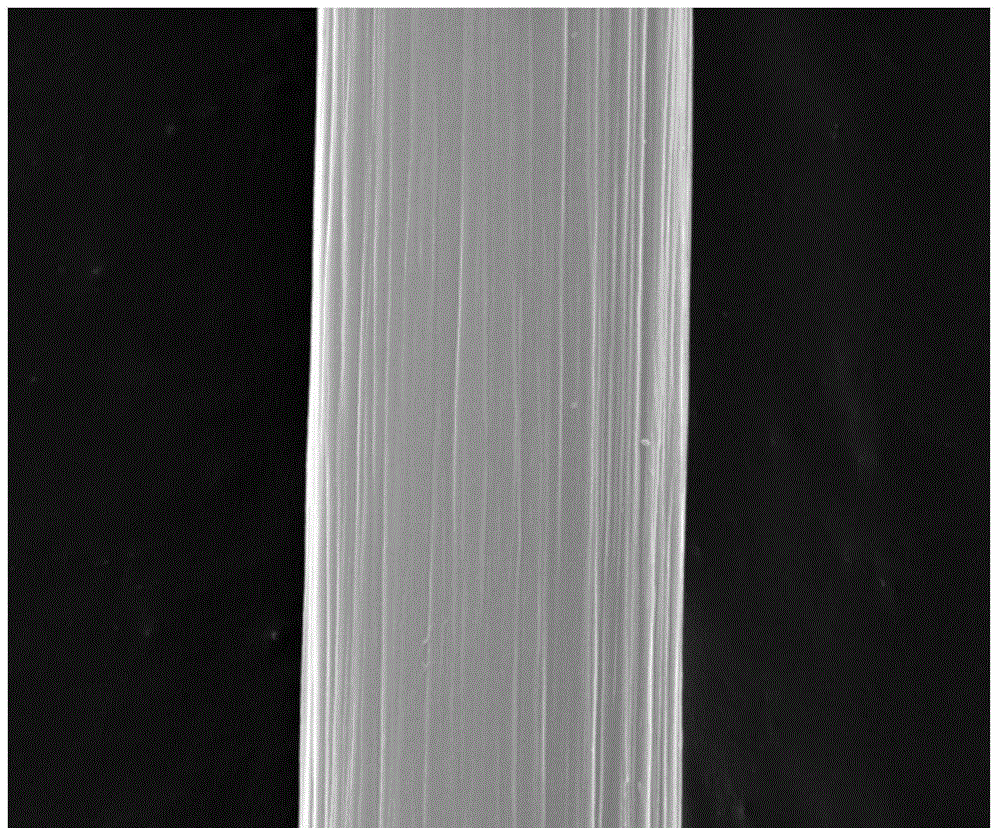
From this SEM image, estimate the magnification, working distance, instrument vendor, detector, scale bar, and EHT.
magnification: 20 K X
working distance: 10 mm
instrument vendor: FEI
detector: SE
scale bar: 5000 nm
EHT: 30 kV